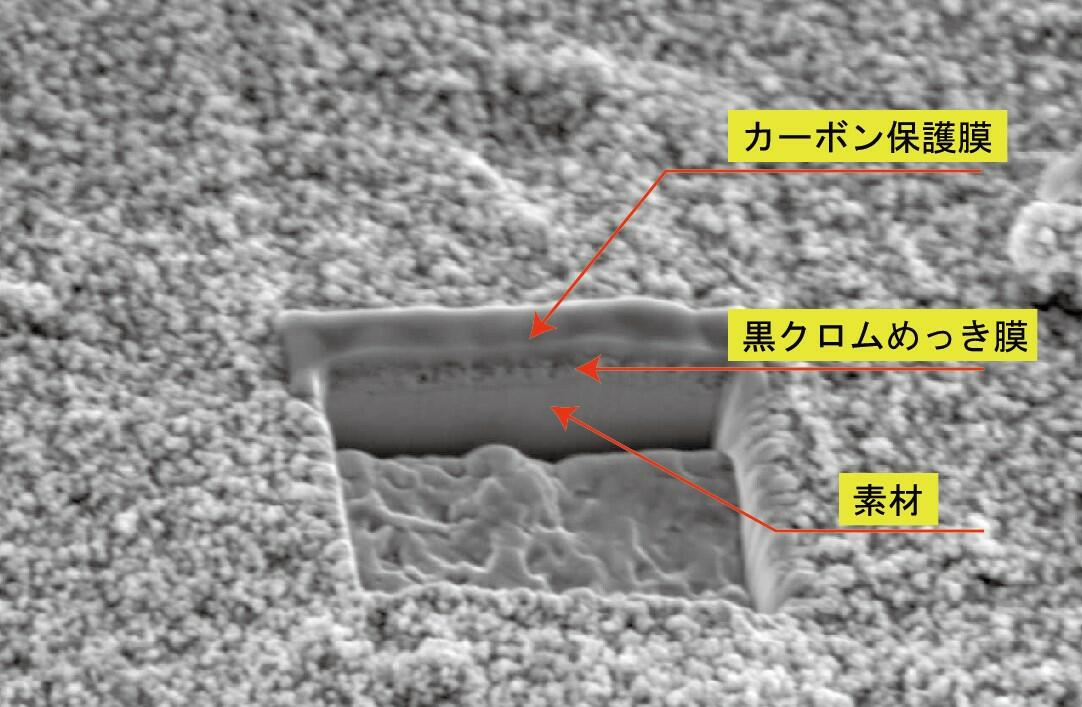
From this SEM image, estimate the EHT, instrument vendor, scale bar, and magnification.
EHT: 10 kV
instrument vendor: JEOL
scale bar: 5000 nm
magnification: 4 K X